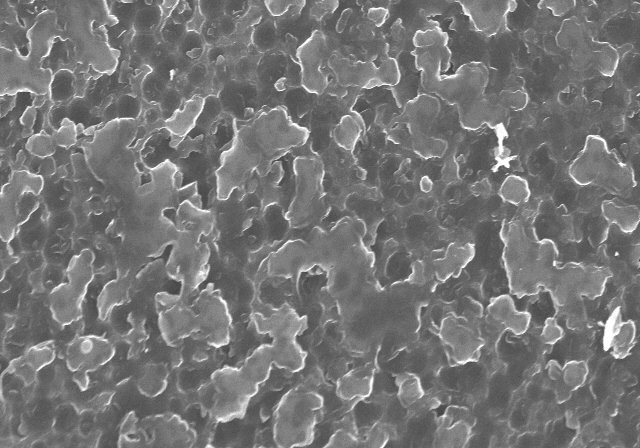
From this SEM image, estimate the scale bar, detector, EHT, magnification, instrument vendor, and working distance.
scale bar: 5000 nm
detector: SE(U)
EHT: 15 kV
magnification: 8.02 K X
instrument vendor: Hitachi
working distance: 9.9 mm